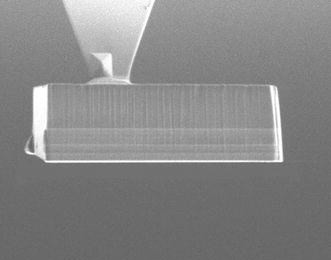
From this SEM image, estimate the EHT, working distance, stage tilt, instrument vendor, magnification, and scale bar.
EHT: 30 kV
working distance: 16.6 mm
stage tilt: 47°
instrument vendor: FEI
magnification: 1.5 K X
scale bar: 30000 nm